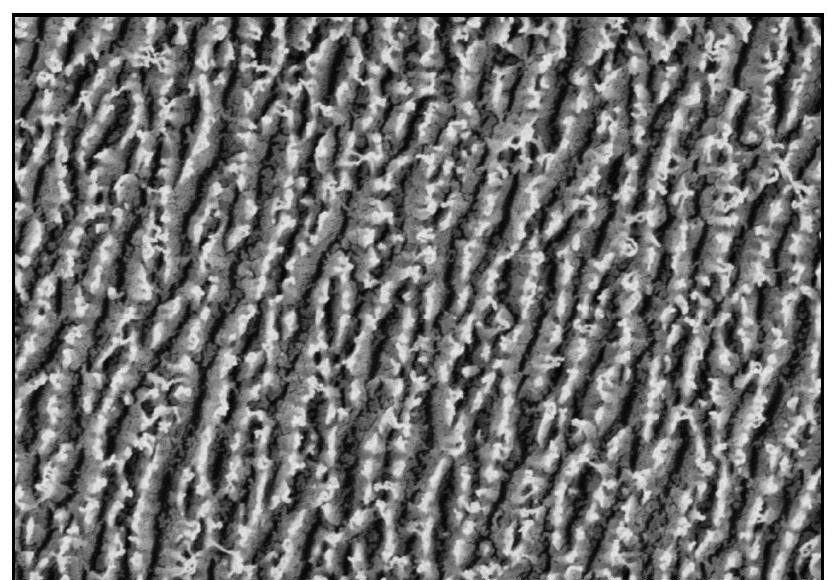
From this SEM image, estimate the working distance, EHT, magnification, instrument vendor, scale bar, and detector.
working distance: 10.4 mm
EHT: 3 kV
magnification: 25 K X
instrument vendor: Hitachi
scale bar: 2000 nm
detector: SE(U)